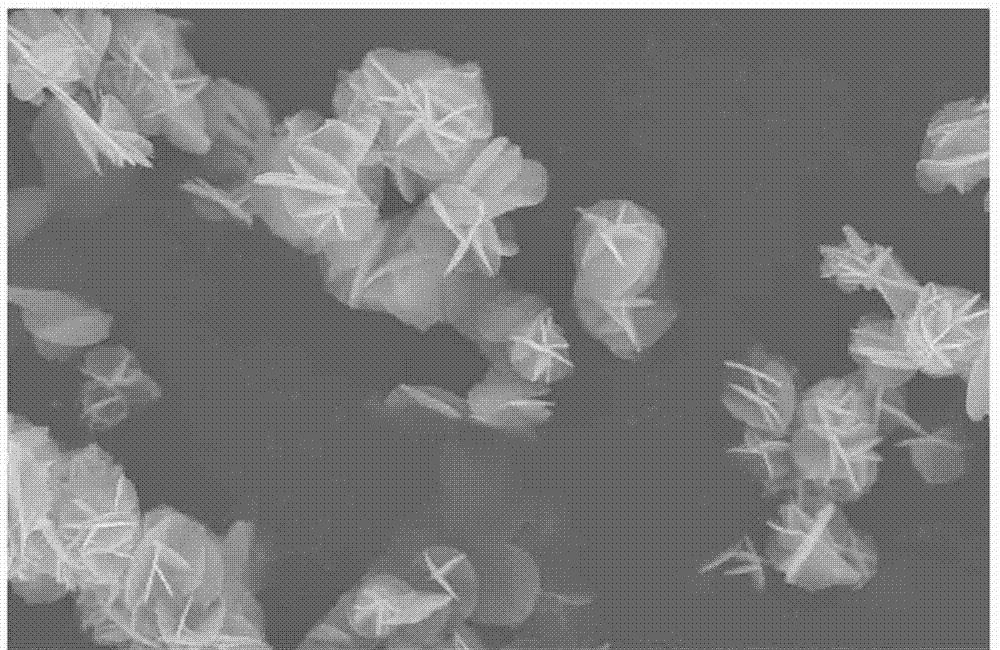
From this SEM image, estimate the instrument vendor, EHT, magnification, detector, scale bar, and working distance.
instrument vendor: Hitachi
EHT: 15 kV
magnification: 10 K X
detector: SE(M)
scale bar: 5000 nm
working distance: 8.7 mm